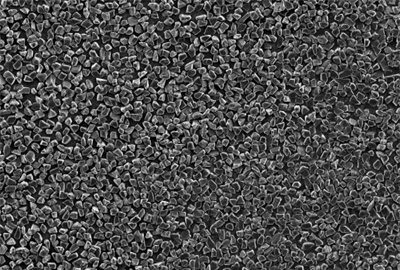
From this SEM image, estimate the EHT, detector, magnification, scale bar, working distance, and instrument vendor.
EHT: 10 kV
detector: SEI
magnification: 0.5 K X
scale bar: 10000 nm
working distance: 10 mm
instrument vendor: JEOL